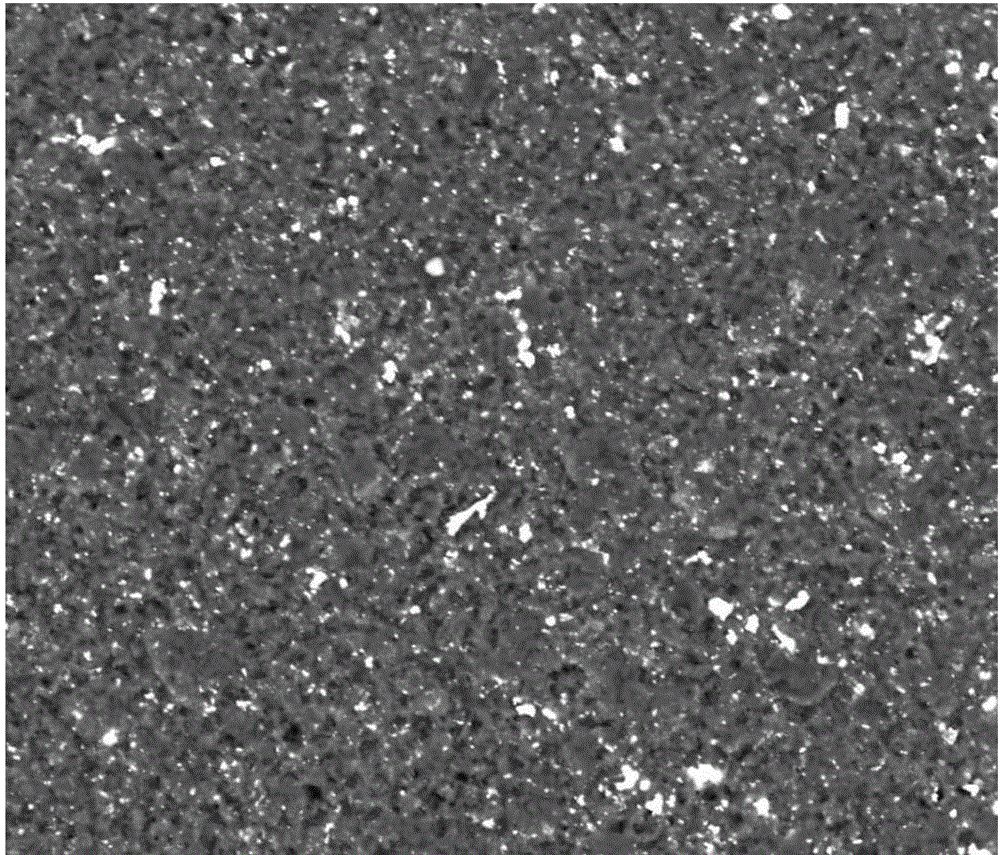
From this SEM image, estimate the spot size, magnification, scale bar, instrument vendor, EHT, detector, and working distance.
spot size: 6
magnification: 2 K X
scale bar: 50000 nm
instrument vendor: FEI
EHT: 20 kV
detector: DualBSD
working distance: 9.8 mm